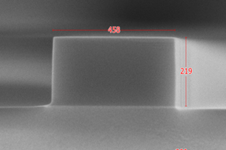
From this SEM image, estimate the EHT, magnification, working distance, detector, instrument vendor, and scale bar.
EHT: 3 kV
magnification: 150 K X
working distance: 0.1 mm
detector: SE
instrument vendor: Hitachi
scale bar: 300 nm